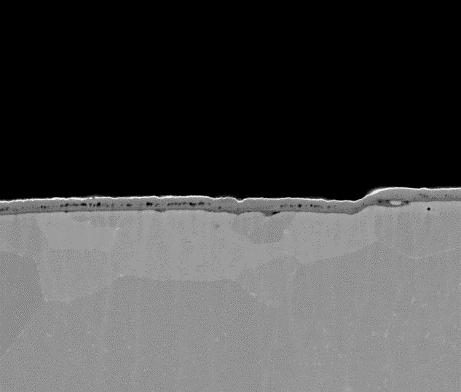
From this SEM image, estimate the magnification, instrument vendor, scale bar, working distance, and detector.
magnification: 5 K X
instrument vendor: FEI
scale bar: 20000 nm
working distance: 10.7 mm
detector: SSD